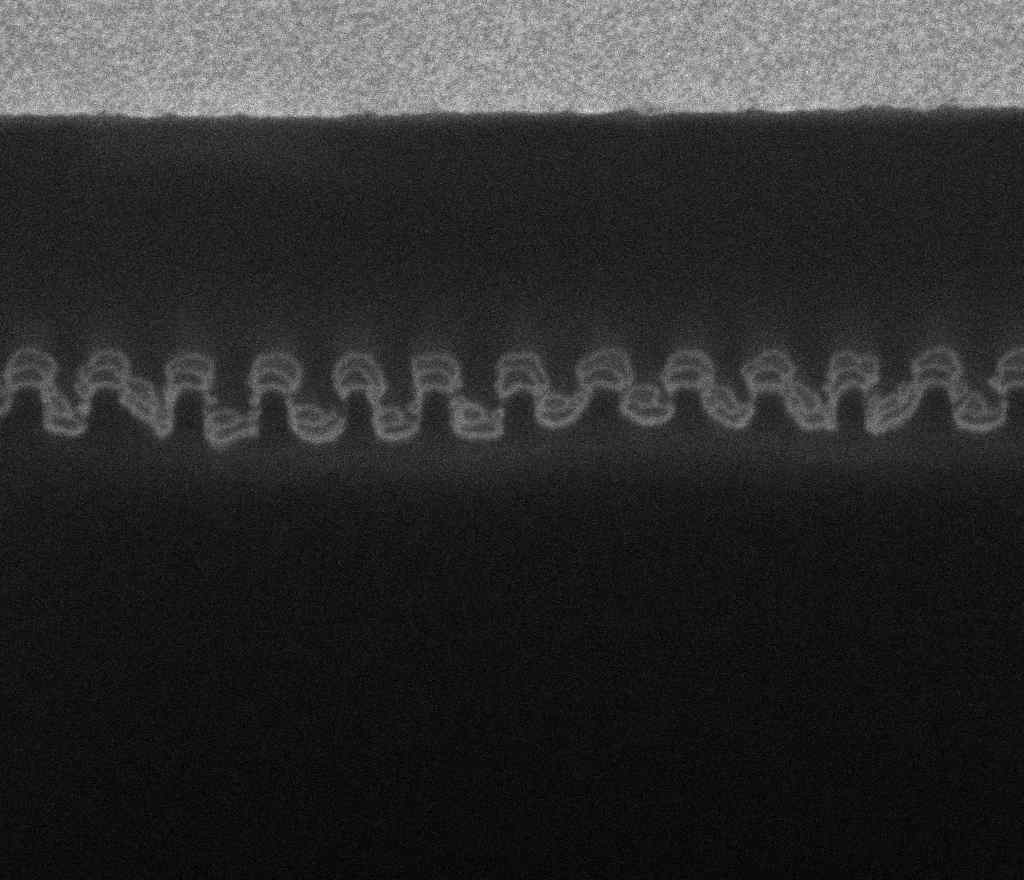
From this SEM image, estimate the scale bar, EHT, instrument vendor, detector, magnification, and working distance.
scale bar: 500 nm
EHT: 5 kV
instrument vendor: FEI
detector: TLD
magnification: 100 K X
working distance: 4.1 mm